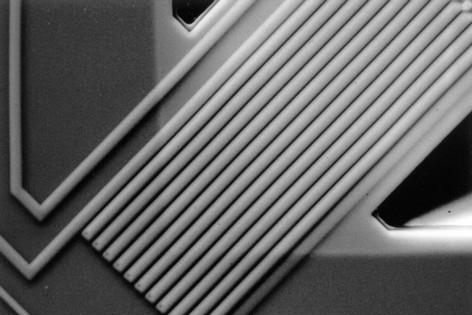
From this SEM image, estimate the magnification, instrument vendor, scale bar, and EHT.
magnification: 0.5 K X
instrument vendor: JEOL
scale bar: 50000 nm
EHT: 30 kV